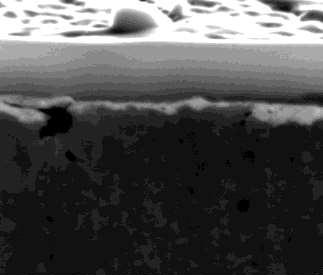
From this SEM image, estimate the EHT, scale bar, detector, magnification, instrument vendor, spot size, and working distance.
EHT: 20 kV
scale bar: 1000 nm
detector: ETD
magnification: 40 K X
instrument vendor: FEI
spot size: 7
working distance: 10 mm